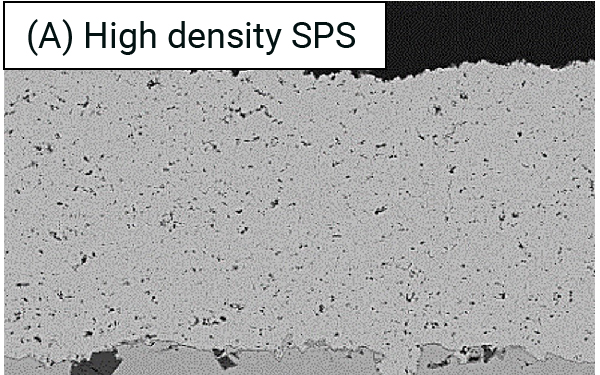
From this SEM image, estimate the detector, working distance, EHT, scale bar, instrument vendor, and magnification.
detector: YAGBSE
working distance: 15.5 mm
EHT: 10 kV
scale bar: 20000 nm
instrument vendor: Hitachi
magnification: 1 K X